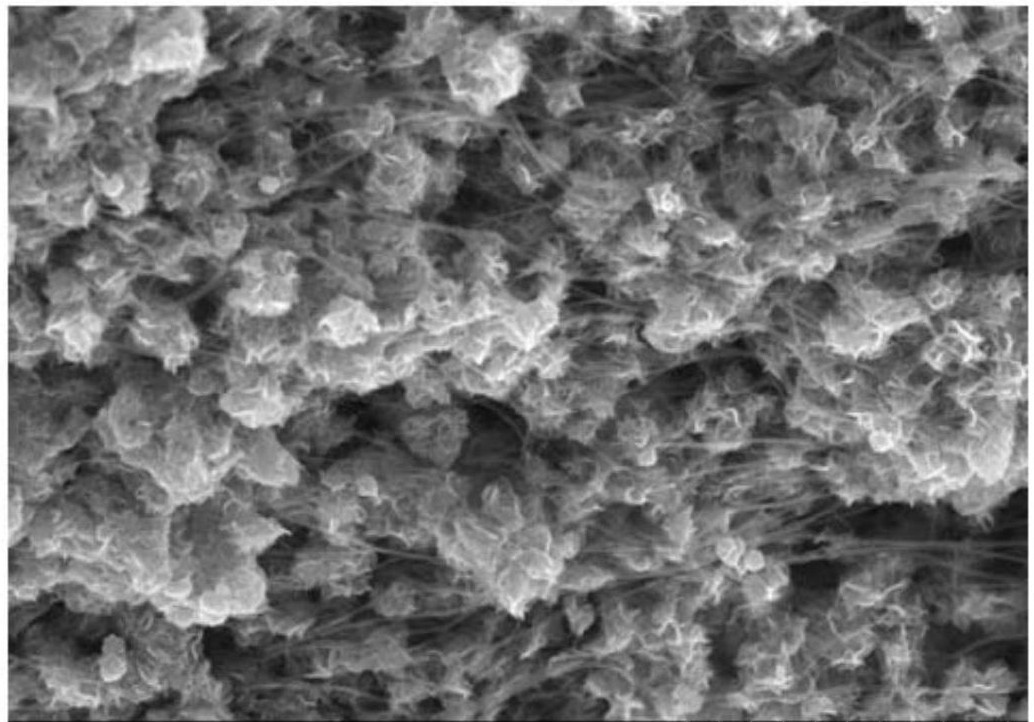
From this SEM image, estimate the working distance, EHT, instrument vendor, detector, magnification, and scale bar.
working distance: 8.2 mm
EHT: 5 kV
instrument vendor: Hitachi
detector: SE(U)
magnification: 20 K X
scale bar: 2000 nm